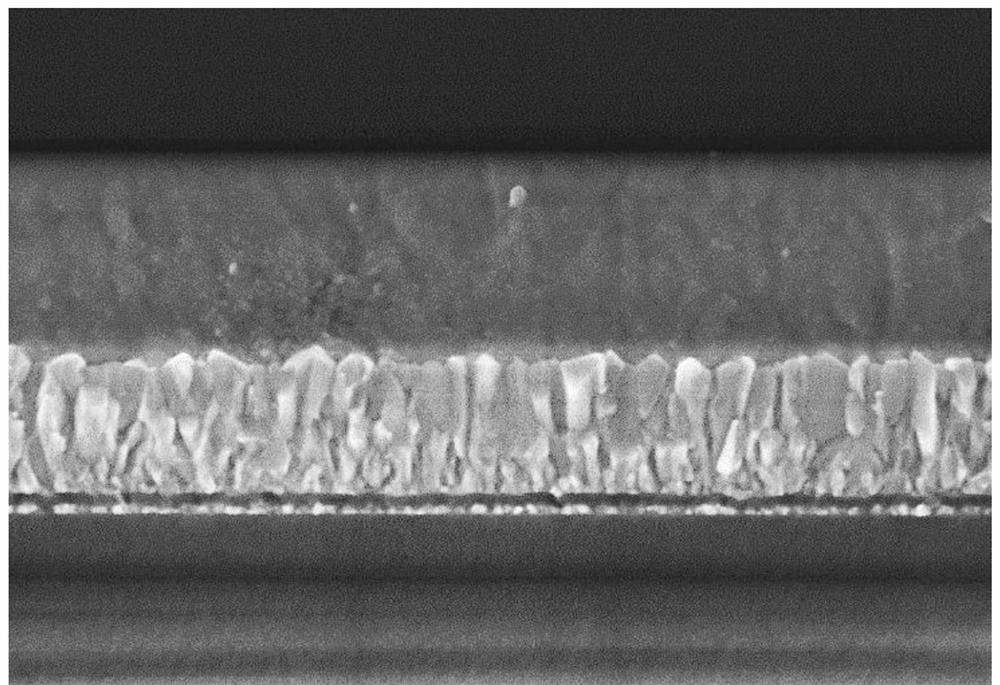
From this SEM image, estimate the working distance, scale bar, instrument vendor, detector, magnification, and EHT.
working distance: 6.7 mm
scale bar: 1000 nm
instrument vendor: Hitachi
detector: SE(U)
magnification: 50 K X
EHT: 3 kV